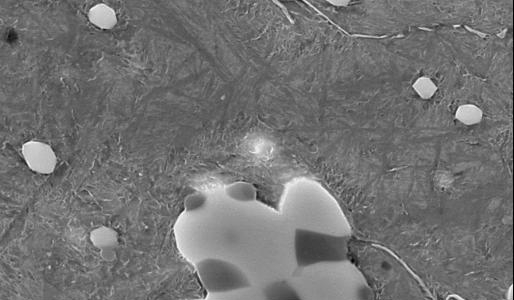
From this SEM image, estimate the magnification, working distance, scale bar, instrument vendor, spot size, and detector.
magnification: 6.5 K X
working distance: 10.7 mm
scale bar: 5000 nm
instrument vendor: FEI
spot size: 4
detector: SE